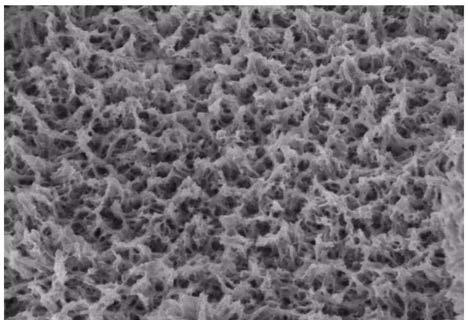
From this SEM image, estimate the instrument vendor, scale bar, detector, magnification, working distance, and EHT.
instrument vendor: Hitachi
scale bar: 2000 nm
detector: SE(UL)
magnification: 20 K X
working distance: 10 mm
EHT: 3 kV